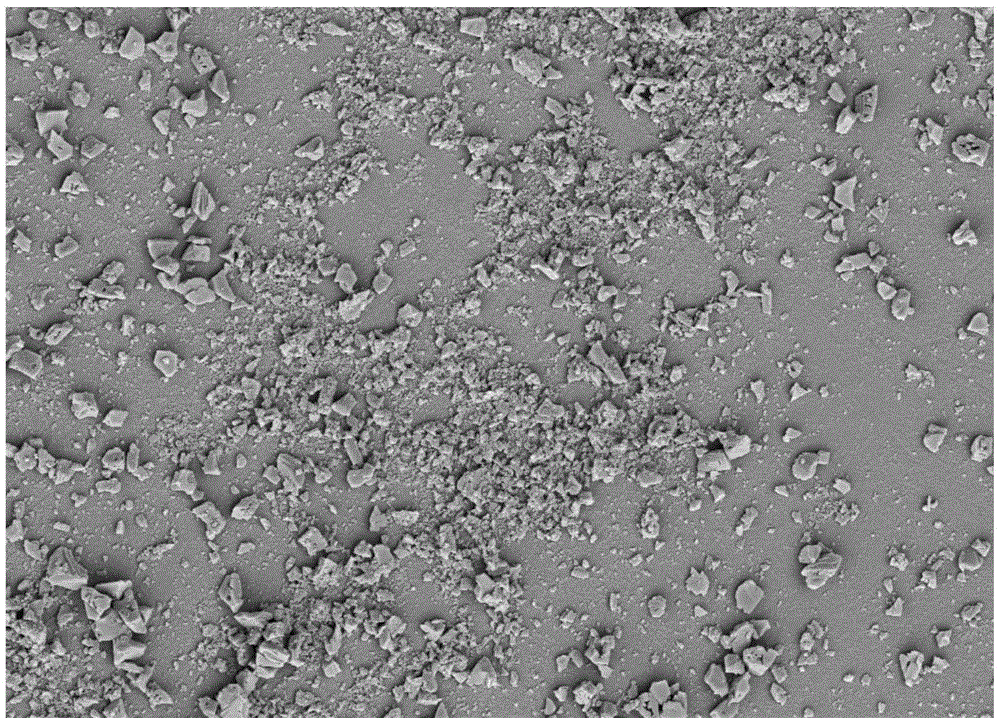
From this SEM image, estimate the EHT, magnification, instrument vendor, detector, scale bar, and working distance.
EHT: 5 kV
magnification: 10 K X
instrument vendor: Zeiss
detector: SE2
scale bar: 1000 nm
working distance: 6.2 mm